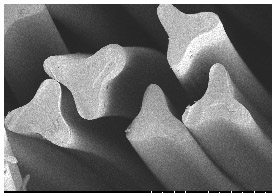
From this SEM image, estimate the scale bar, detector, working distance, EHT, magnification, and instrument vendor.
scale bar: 30000 nm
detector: SE(M)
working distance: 11.4 mm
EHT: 1 kV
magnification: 1.8 K X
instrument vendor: Hitachi